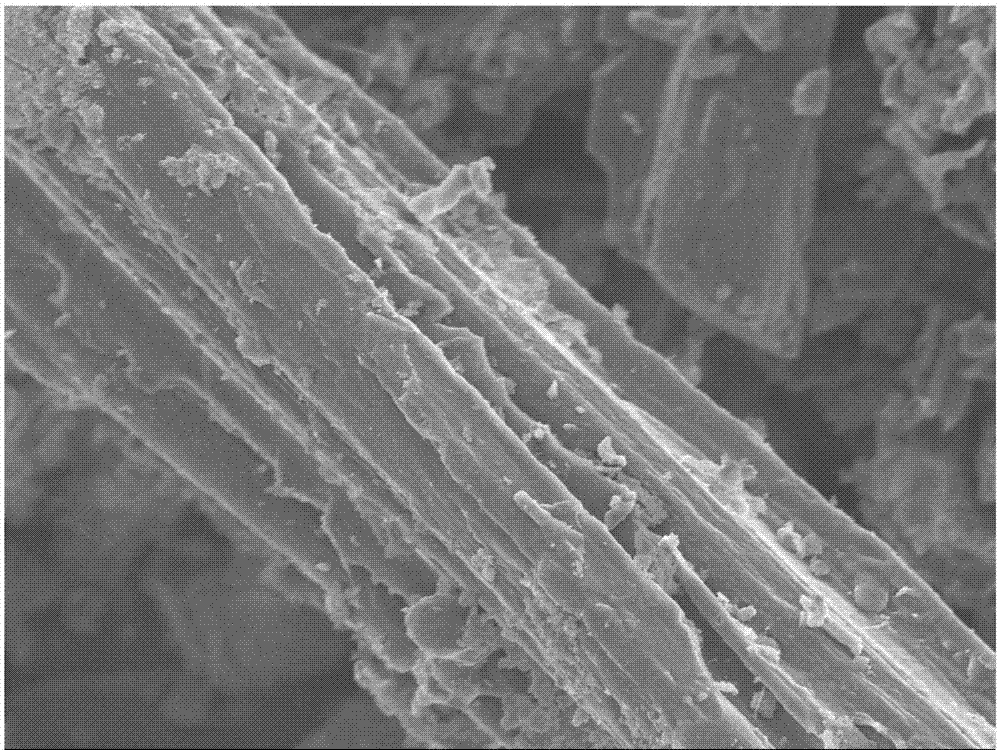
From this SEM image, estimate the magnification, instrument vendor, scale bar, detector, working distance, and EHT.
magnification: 3 K X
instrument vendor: JEOL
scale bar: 1000 nm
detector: SEI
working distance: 7.5 mm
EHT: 5 kV